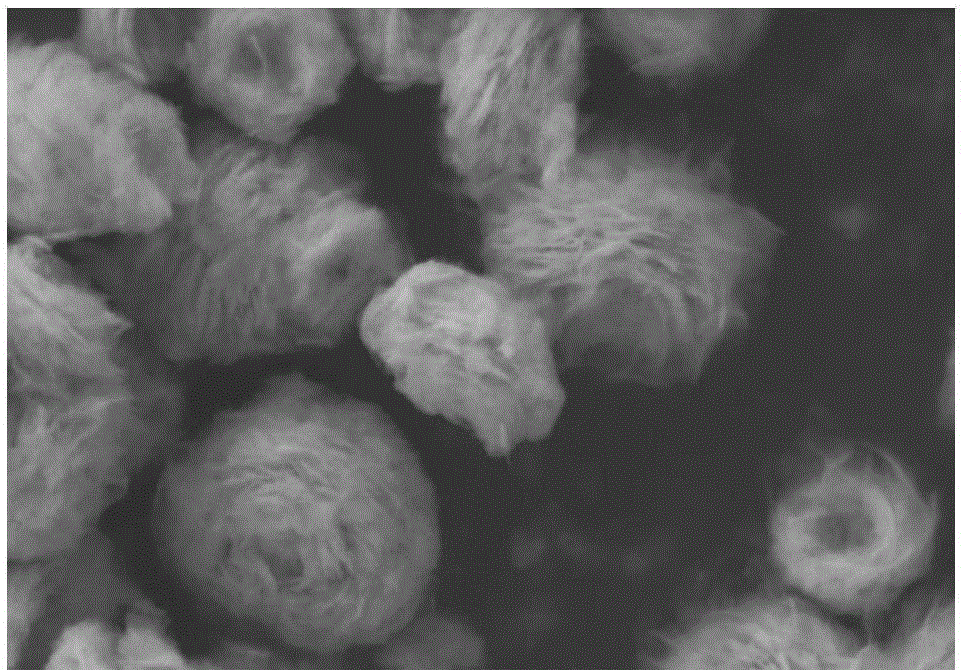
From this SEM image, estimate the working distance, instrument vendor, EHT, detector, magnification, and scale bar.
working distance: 6.6 mm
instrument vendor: Zeiss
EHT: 5 kV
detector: SESI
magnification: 28.2 K X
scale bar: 300 nm